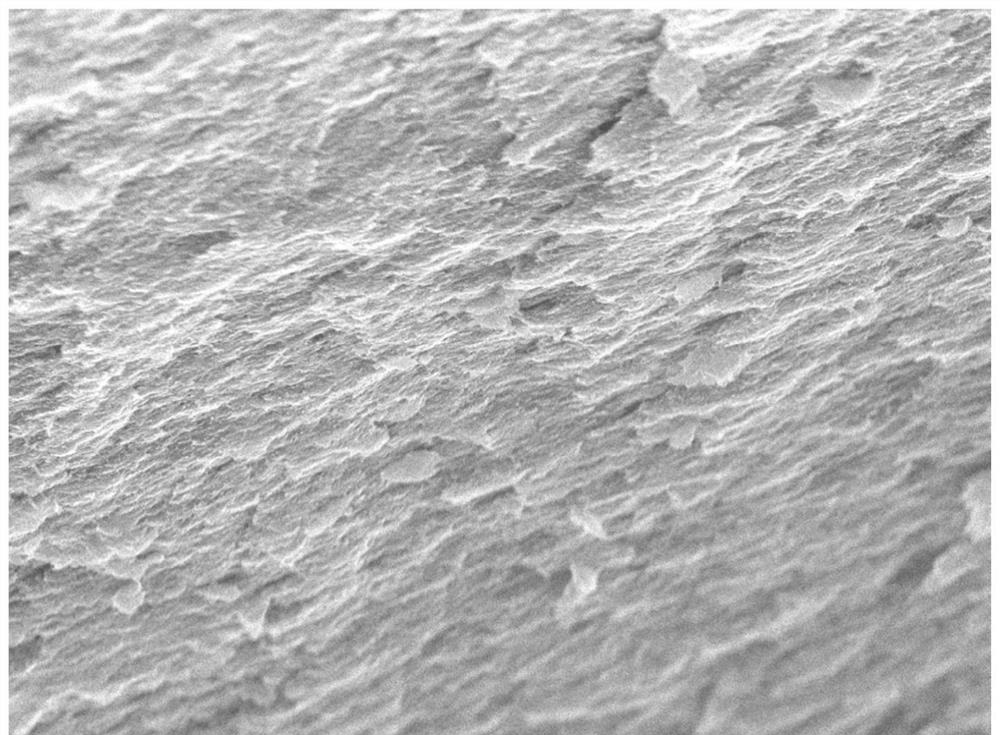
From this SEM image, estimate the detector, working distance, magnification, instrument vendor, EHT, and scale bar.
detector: SEI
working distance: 6 mm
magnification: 9.5 K X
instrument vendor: JEOL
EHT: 15 kV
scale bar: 1000 nm